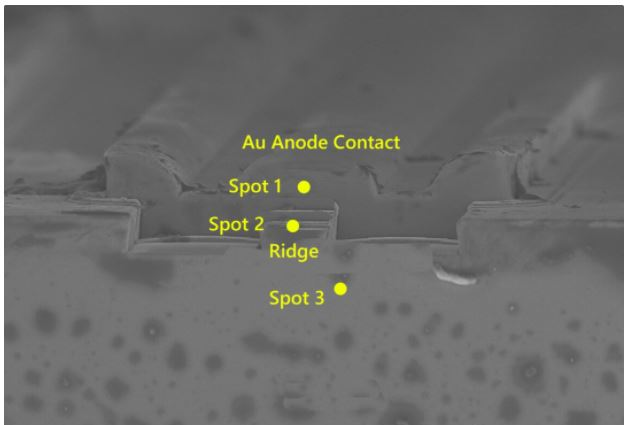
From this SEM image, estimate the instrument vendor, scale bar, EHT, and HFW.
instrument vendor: Zeiss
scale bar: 2000 nm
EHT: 5 kV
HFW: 36.47 µm